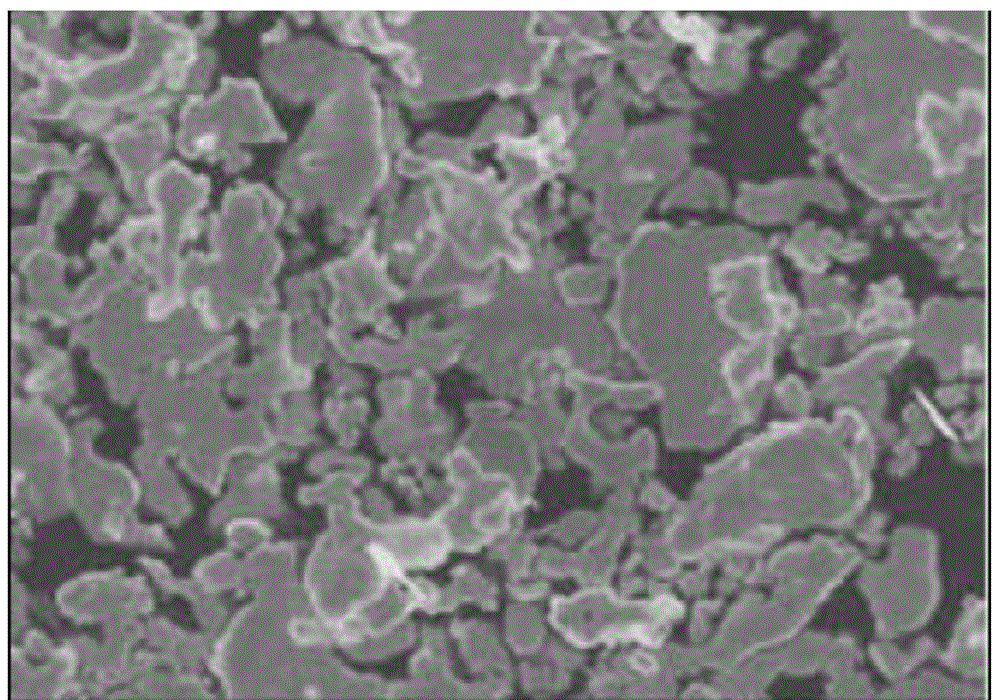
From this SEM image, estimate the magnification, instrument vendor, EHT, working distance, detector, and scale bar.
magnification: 5 K X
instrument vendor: JEOL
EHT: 10 kV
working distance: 8 mm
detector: SEI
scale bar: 1000 nm